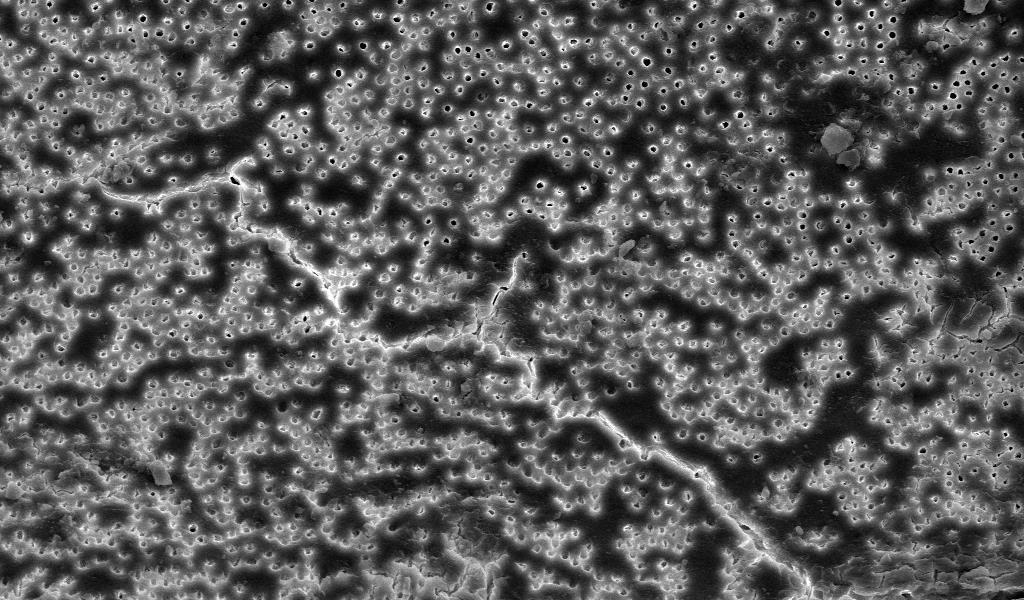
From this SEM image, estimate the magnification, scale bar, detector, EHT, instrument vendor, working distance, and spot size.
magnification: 1 K X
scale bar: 100000 nm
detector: ETD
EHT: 20 kV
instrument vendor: FEI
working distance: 19.5 mm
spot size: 3